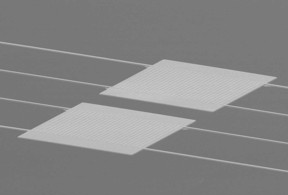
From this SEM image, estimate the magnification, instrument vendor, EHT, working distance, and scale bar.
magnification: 2.5 K X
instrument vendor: Hitachi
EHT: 10 kV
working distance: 11.3 mm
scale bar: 50000 nm